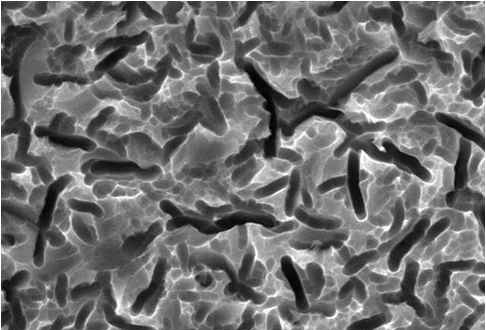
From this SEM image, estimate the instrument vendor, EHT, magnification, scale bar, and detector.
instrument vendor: JEOL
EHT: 20 kV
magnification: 3 K X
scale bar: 5000 nm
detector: SEI